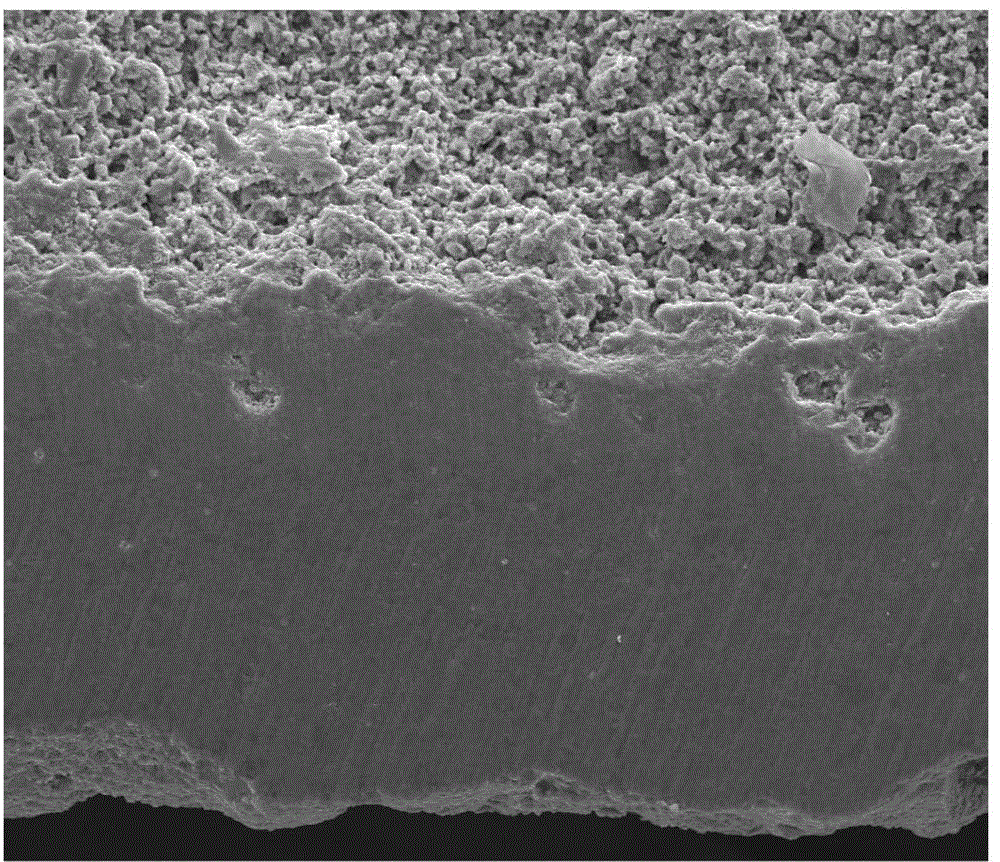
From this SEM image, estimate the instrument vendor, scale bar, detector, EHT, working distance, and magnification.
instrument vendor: FEI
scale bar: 100000 nm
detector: ETD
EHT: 20 kV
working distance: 11.2 mm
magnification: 1 K X